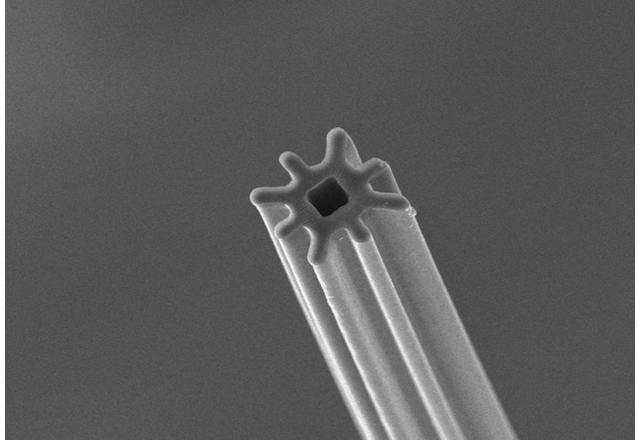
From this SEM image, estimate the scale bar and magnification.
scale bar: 10000 nm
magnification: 1 K X